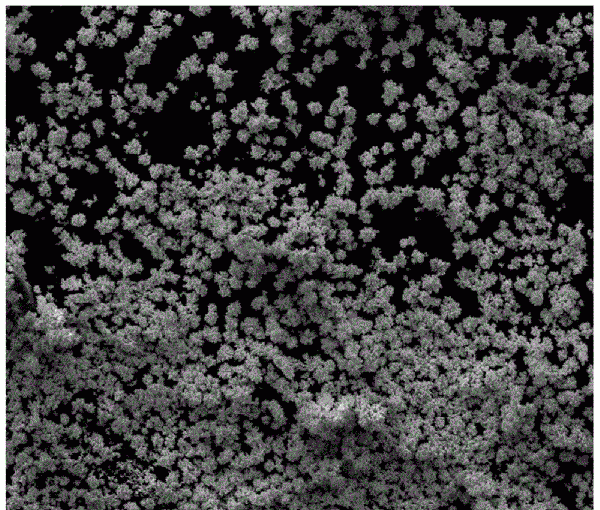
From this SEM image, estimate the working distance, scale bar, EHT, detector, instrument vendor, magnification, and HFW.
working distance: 11 mm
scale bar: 200000 nm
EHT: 20 kV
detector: ETD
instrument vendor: FEI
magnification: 0.24 K X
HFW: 529 µm